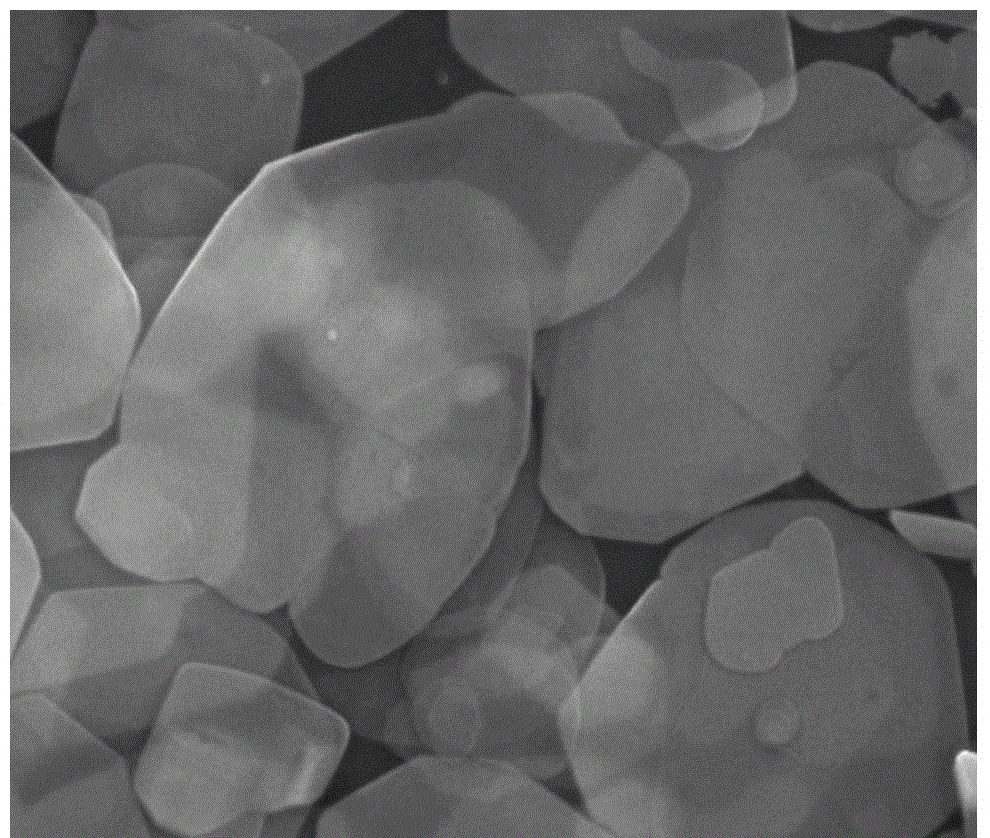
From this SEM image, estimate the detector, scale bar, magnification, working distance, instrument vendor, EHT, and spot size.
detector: ETD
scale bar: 2000 nm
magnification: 50 K X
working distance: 5.2 mm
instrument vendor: FEI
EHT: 15 kV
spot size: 3